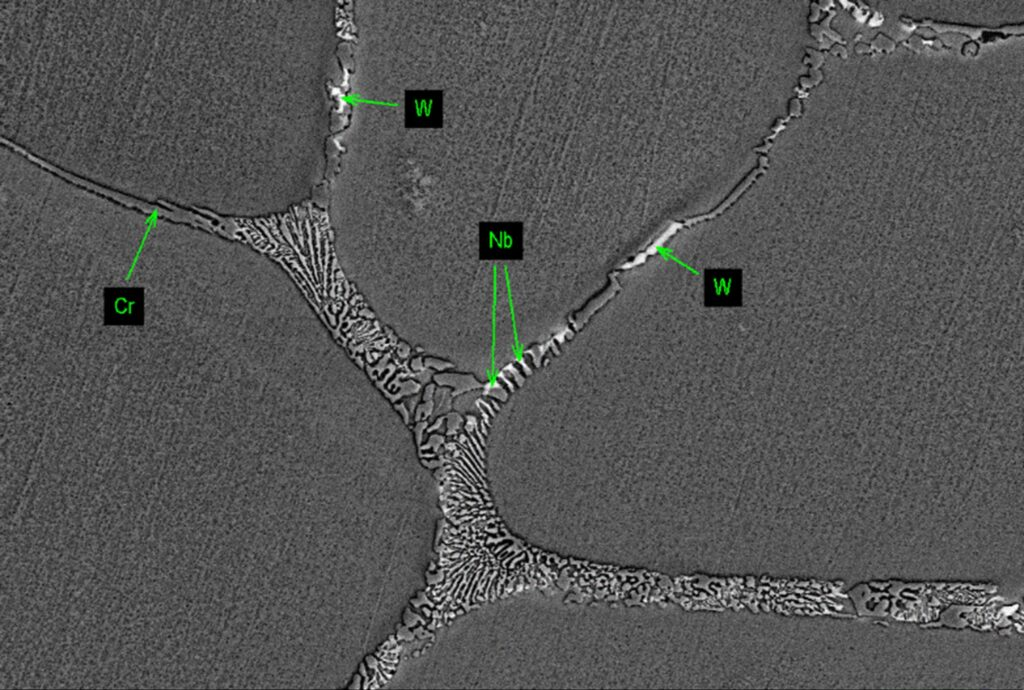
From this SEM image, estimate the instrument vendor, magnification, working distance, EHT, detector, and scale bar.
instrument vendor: Zeiss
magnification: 1 K X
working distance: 8.5 mm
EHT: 20 kV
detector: AsB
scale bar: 10000 nm